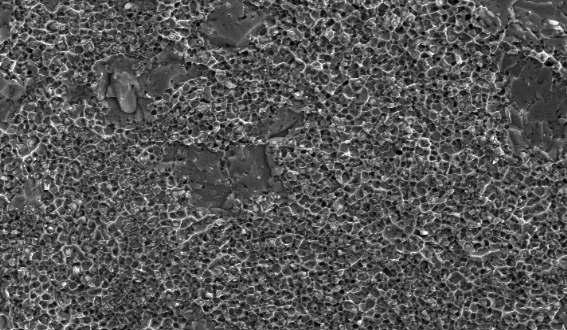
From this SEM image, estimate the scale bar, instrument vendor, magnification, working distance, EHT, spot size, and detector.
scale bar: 5000 nm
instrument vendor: FEI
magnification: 10 K X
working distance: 5 mm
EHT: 10 kV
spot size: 3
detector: SE